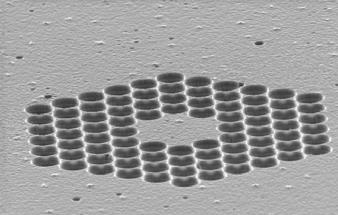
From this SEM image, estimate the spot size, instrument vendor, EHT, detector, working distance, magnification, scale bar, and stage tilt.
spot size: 3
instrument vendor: FEI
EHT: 15 kV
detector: SE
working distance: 19.2 mm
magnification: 1.149 K X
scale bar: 20000 nm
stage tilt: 0°